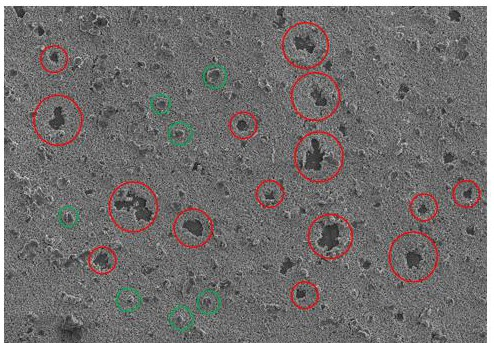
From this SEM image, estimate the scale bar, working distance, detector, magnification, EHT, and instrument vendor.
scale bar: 100000 nm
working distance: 10.6 mm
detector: SE(L)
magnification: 0.5 K X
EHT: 5 kV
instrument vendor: Hitachi